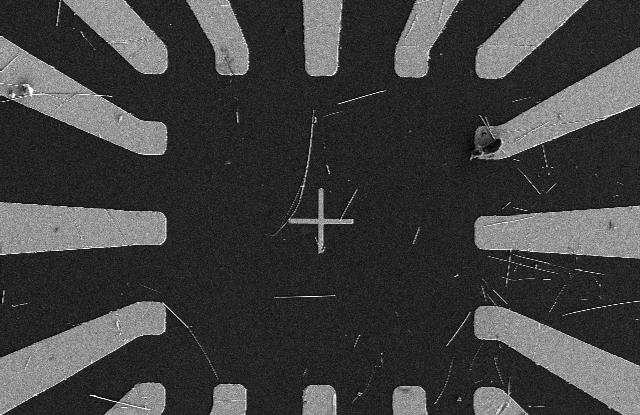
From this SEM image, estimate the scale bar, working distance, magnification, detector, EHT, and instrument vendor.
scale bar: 50000 nm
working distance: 8.8 mm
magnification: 0.9 K X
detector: SE(L)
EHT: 5 kV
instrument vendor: Hitachi